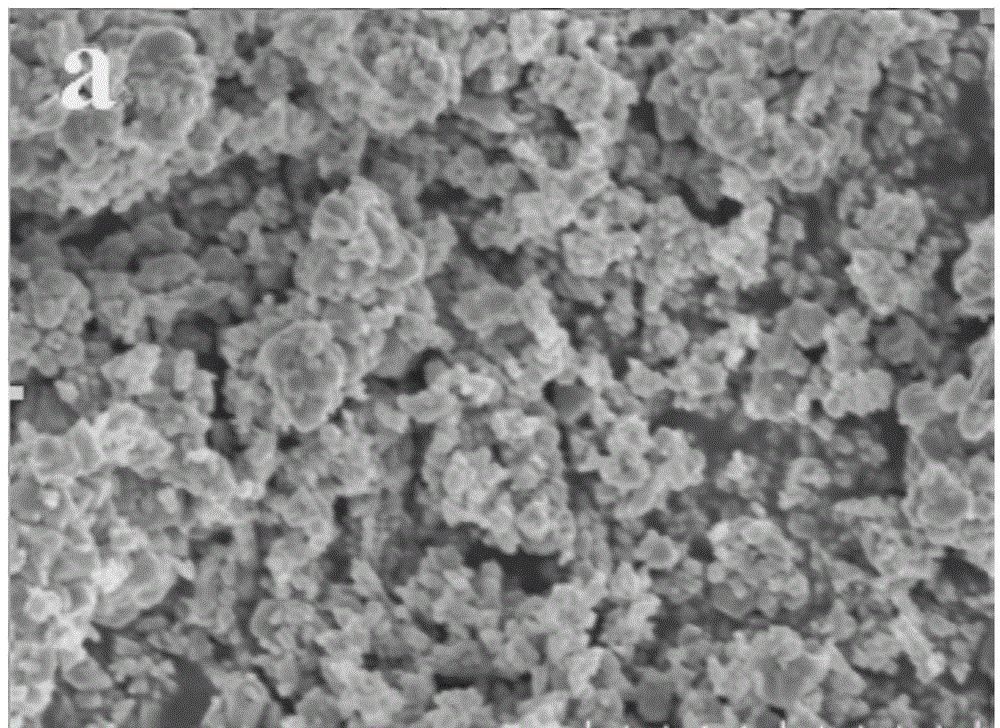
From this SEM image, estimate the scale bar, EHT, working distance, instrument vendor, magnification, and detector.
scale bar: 5000 nm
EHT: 5 kV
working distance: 8.8 mm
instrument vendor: Hitachi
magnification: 10 K X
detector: SE(UL)